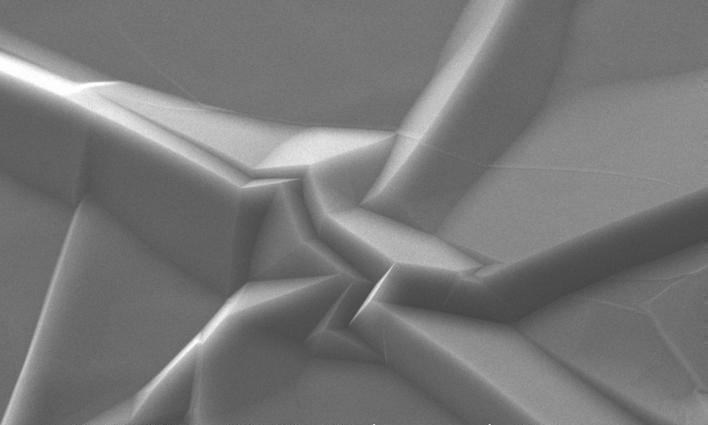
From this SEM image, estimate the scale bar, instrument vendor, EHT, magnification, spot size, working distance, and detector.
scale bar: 10000 nm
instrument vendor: FEI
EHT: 20 kV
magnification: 1.889 K X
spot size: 3.1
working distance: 3.7 mm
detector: SE